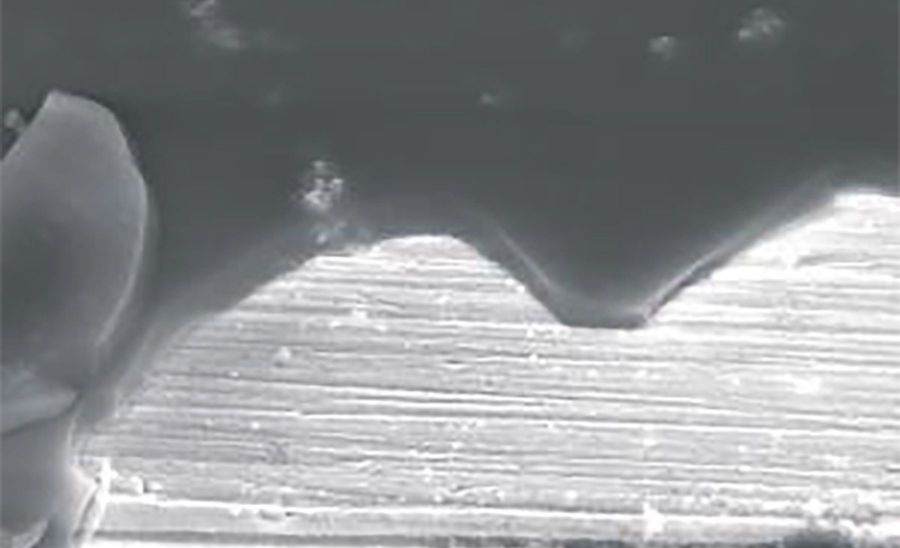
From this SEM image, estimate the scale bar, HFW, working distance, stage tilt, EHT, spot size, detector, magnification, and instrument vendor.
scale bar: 4000 nm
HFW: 20.7 µm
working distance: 6.7 mm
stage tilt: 45°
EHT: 15 kV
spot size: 4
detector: ETD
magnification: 20 K X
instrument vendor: FEI